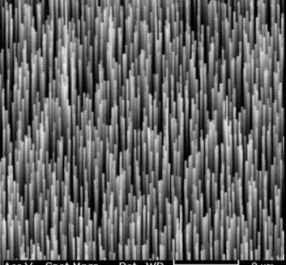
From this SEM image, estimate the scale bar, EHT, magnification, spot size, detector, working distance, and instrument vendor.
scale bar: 2000 nm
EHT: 15 kV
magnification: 8.902 K X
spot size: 4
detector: SE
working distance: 11.2 mm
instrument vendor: FEI